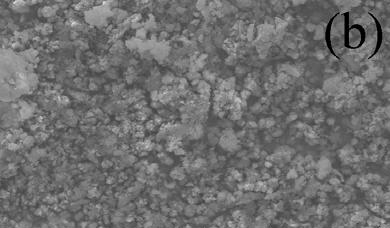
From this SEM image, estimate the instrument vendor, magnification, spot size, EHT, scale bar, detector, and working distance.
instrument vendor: FEI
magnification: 1 K X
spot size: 3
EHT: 20 kV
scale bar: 20000 nm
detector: SE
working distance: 10.3 mm